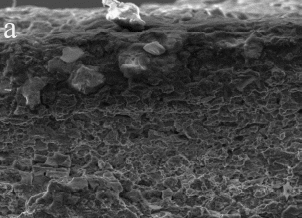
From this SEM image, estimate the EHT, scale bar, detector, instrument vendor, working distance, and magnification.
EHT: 20 kV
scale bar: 100000 nm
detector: ETD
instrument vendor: FEI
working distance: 12.7 mm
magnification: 1 K X